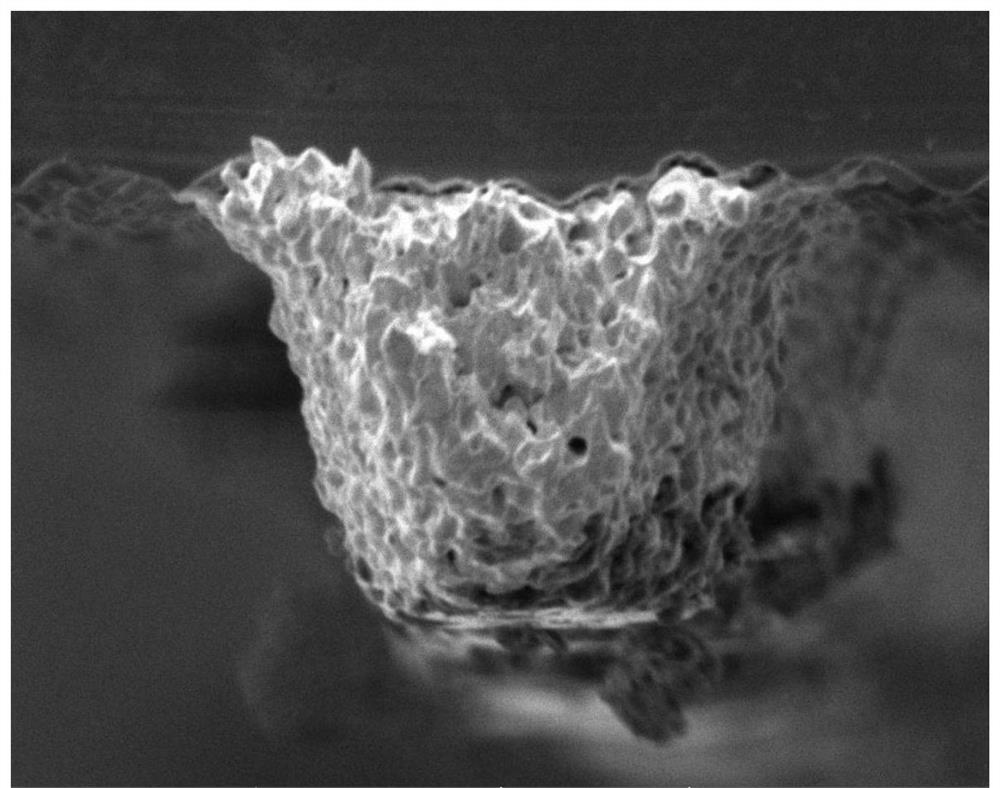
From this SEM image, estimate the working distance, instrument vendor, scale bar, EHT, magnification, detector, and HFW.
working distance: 17.86 mm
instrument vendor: Tescan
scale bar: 10000 nm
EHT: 5 kV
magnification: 5.33 K X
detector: SE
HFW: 51.9 µm